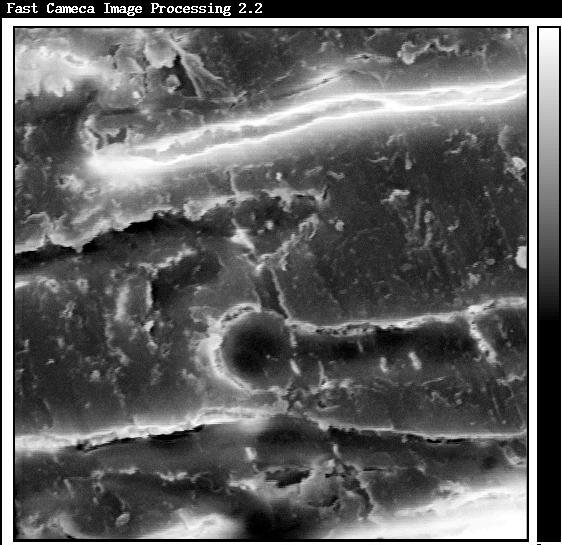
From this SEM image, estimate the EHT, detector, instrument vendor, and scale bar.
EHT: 15 kV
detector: SE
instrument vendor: other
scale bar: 5000 nm